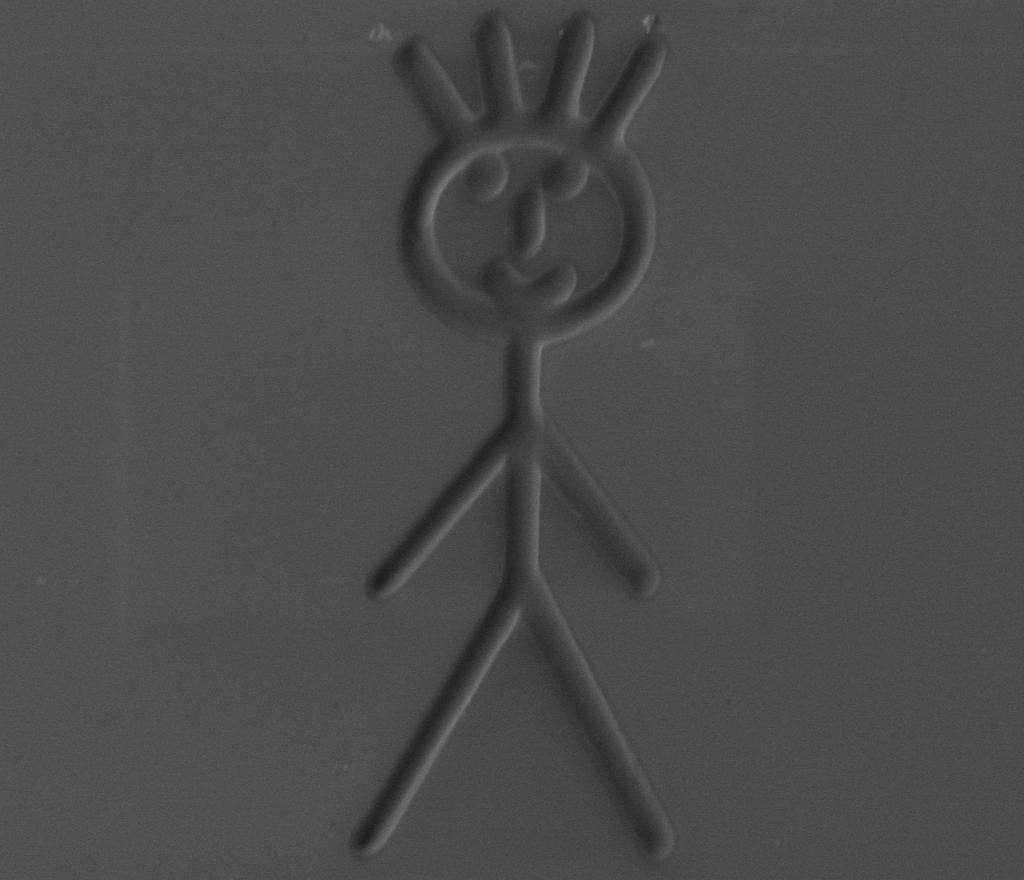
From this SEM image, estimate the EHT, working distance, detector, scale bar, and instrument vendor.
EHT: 10 kV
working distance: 10 mm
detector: CDEM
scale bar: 10000 nm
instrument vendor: FEI